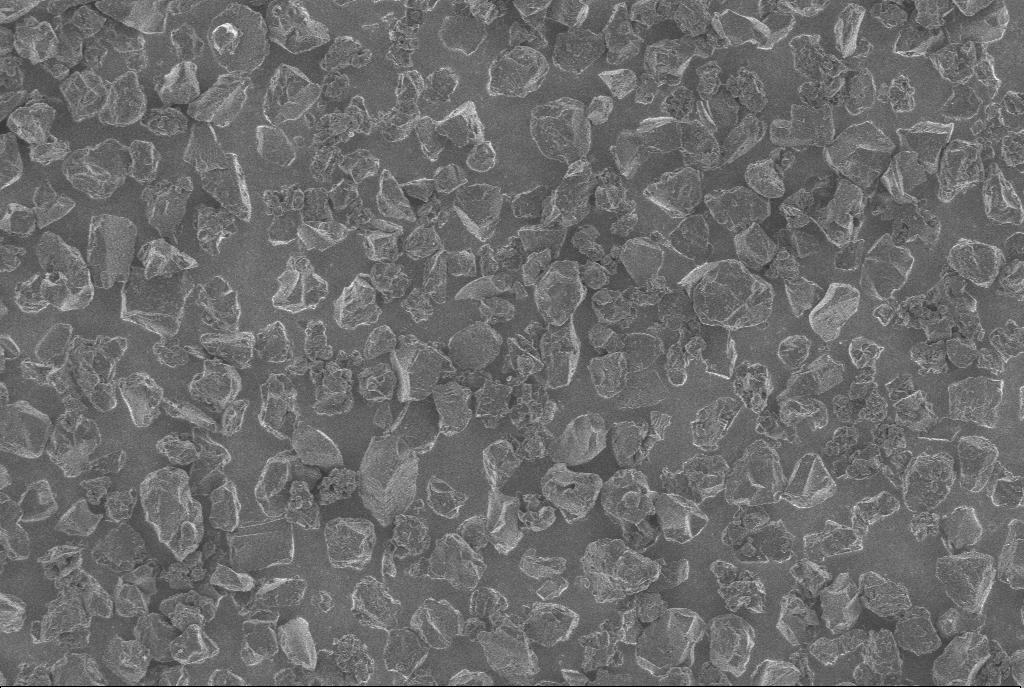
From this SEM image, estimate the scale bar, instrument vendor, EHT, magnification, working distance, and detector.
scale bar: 10000 nm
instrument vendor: Zeiss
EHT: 1 kV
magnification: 1 K X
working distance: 5.6 mm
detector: InLens